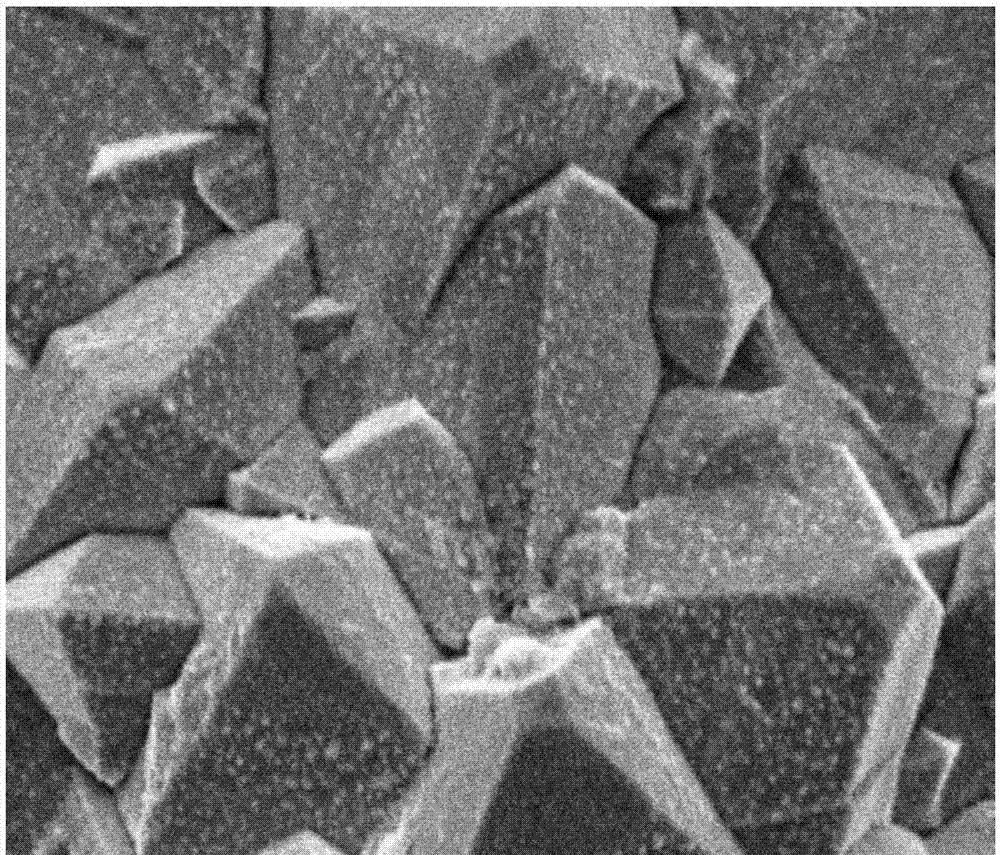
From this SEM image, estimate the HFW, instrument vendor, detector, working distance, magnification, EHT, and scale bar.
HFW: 19.9 µm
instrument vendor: FEI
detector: ETD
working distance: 17.4 mm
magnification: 10 K X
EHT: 10 kV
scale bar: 5000 nm